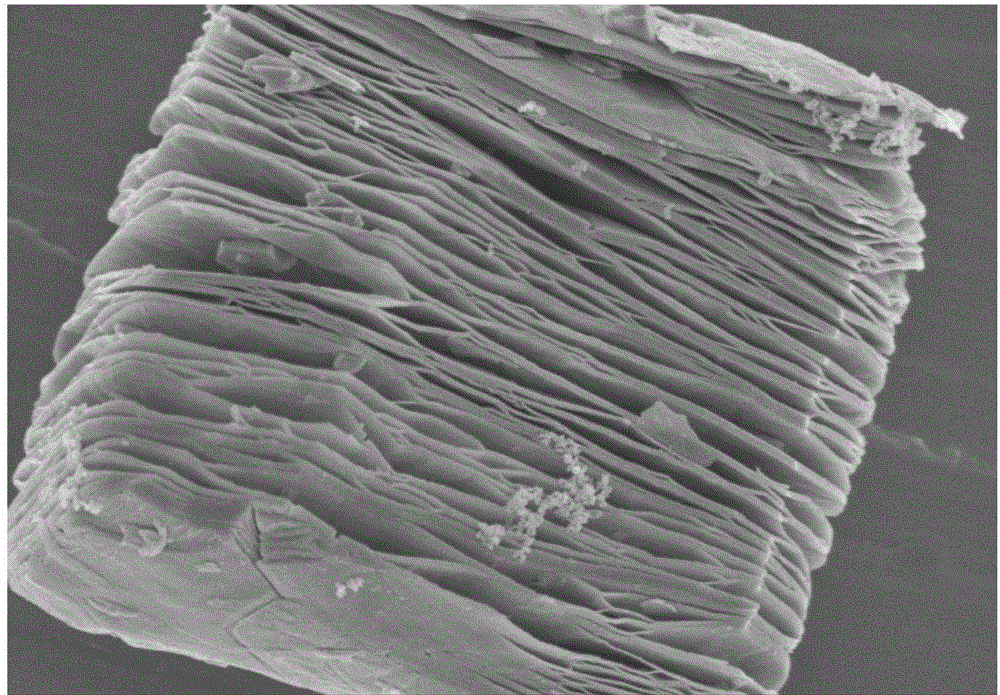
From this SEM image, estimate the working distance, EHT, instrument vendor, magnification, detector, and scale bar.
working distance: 9.2 mm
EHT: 3 kV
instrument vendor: Hitachi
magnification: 20 K X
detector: SE(M)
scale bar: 2000 nm